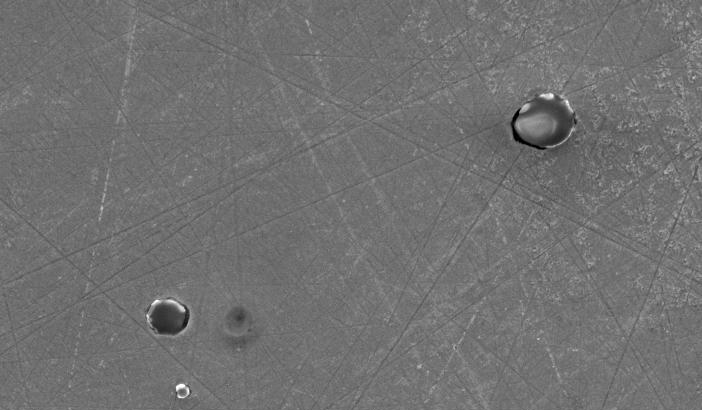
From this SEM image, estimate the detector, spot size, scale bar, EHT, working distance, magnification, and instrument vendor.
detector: SE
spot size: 4.5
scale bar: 20000 nm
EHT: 20 kV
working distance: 11.2 mm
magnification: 1 K X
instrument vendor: FEI